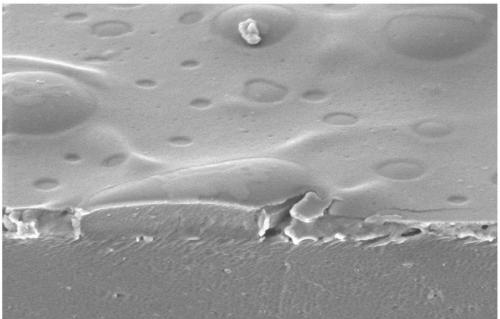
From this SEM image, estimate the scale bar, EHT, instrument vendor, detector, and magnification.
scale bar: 5000 nm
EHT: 3 kV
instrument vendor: Hitachi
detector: SE(M)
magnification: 10 K X